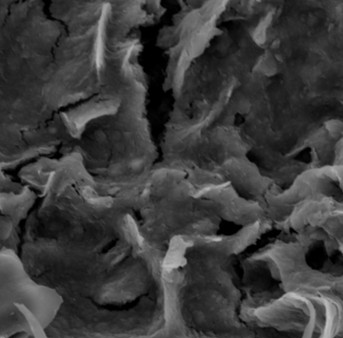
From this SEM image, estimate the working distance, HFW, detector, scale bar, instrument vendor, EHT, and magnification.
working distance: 31.81 mm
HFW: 95.7 µm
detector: SE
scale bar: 20000 nm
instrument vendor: Tescan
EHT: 20 kV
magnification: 1.51 K X